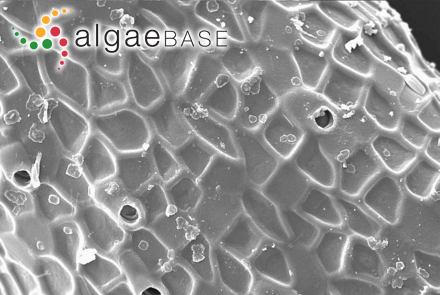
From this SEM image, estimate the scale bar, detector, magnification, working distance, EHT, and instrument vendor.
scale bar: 10000 nm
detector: SE1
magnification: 0.835 K X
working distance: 13 mm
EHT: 13.45 kV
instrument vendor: Zeiss